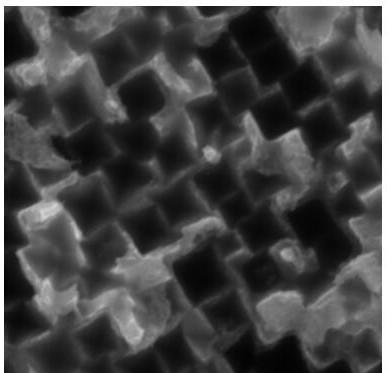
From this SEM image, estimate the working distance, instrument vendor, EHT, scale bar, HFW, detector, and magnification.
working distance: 15.2 mm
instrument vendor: Tescan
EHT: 20 kV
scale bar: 2000 nm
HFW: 10.4 µm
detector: SE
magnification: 20 K X